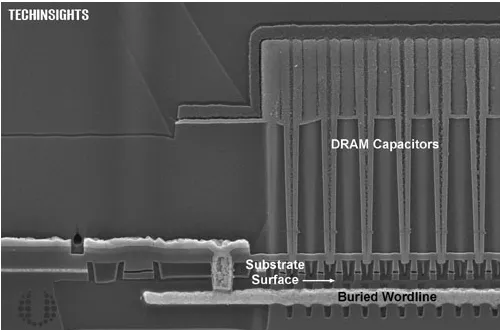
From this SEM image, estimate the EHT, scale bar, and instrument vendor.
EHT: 5 kV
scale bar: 200 nm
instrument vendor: Zeiss